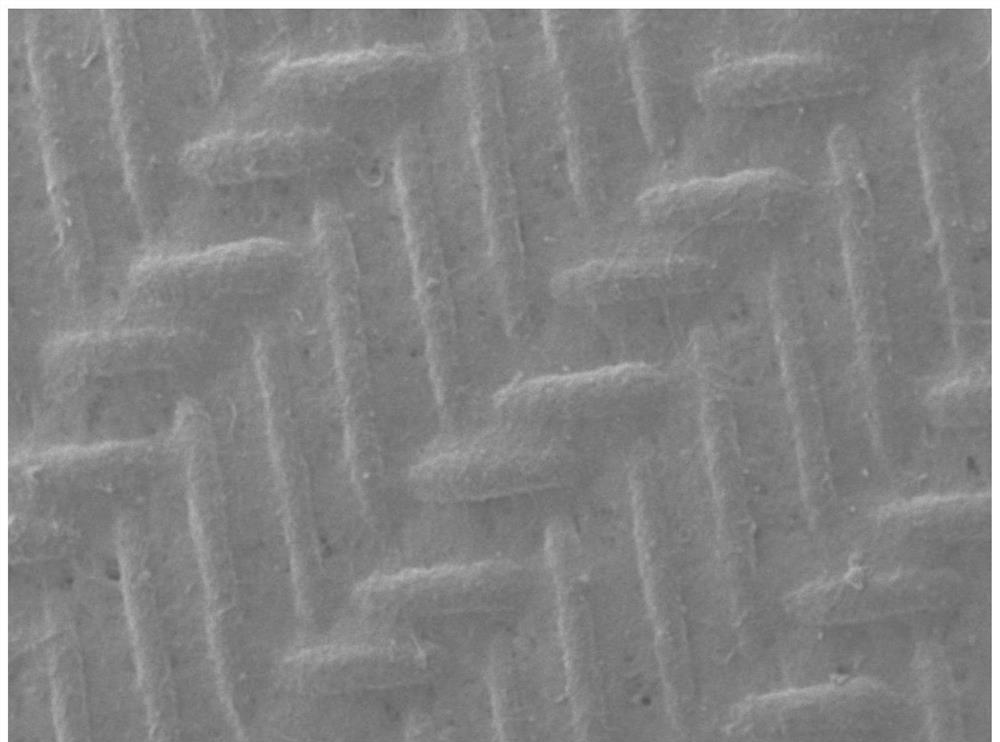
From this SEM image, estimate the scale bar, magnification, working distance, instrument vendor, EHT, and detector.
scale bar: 100000 nm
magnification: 0.1 K X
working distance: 10.4 mm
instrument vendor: JEOL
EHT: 15 kV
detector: SED